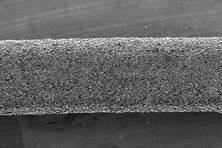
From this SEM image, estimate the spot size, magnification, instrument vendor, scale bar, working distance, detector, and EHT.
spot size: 40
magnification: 0.05 K X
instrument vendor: JEOL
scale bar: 500000 nm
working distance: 18 mm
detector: SEI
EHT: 1.5 kV